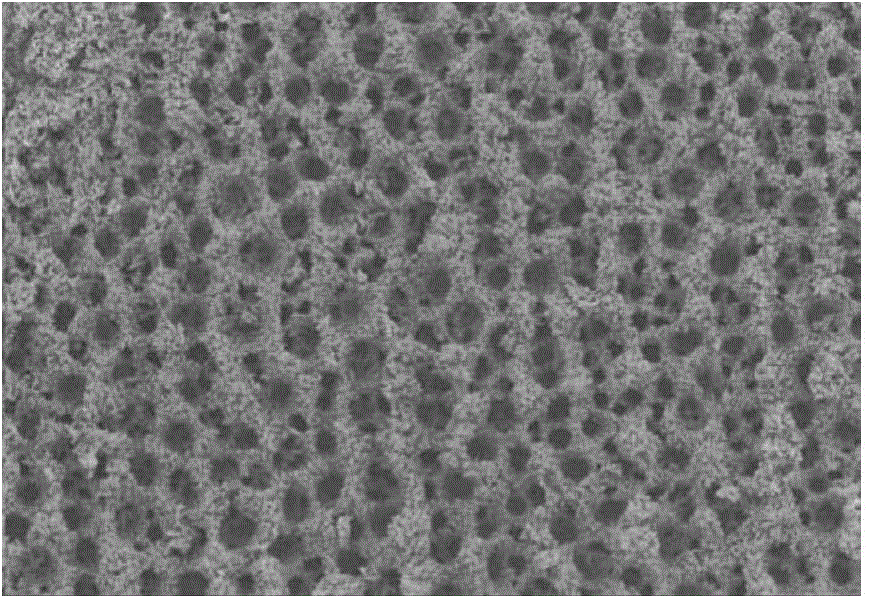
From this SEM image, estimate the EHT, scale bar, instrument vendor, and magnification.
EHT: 15 kV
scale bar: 1000000 nm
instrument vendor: JEOL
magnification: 0.05 K X